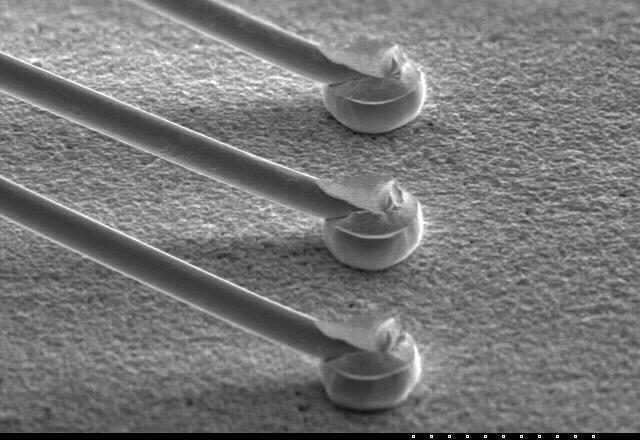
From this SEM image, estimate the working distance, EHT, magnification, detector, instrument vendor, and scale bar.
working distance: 27.5 mm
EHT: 25 kV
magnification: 0.18 K X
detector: SE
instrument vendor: Hitachi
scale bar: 200000 nm